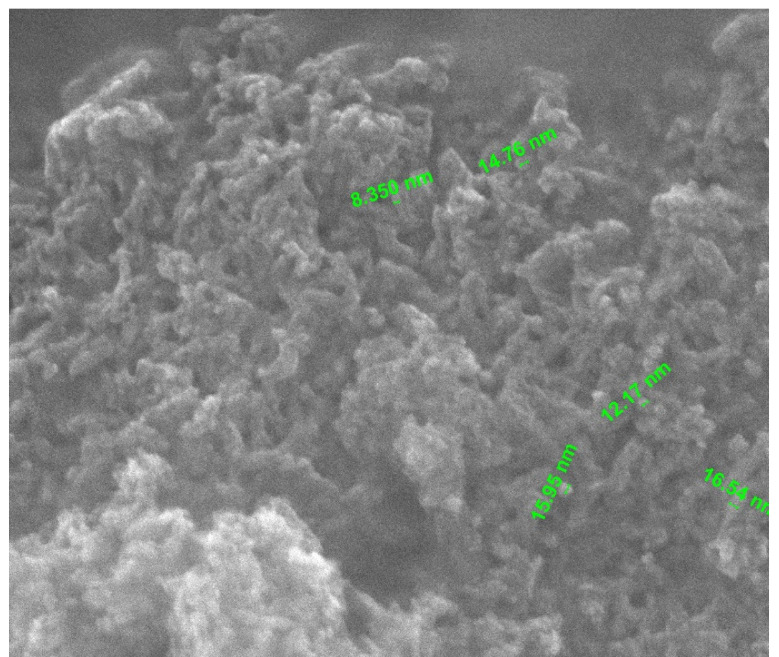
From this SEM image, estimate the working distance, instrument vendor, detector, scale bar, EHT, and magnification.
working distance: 10.1 mm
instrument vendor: FEI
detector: ETD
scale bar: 500 nm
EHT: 30 kV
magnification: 200 K X